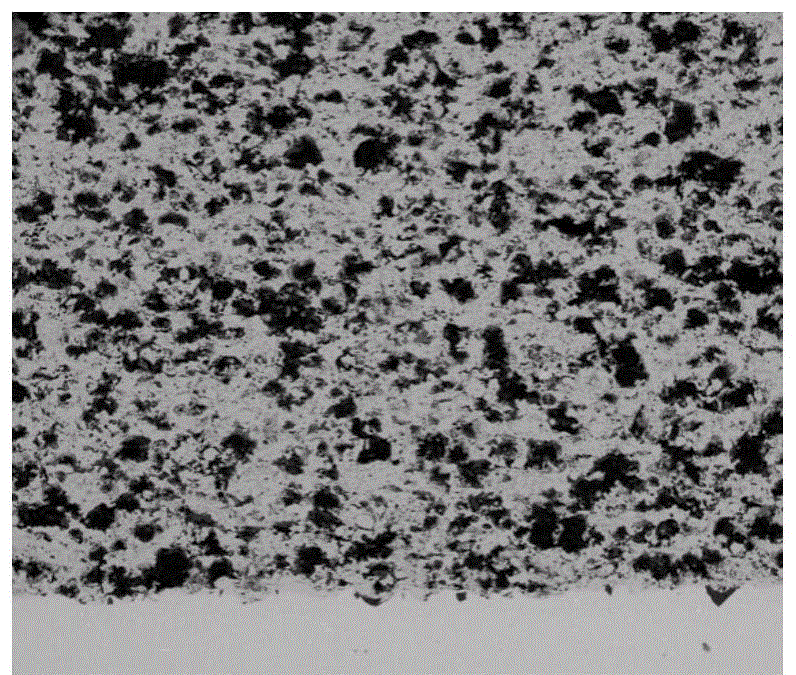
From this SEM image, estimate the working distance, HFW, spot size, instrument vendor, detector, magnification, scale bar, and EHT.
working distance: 18.4 mm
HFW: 2500 µm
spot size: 6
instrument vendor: FEI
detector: DualBSD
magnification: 0.06 K X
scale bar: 500000 nm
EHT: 25 kV